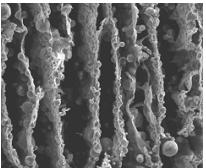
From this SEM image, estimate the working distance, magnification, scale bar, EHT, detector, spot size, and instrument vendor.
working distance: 12.5 mm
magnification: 0.2 K X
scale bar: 400000 nm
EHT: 20 kV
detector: ETD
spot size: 4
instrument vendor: FEI